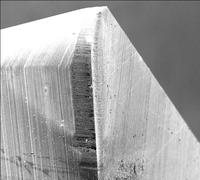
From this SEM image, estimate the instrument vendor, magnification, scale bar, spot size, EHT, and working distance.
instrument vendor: FEI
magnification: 0.093 K X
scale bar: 500000 nm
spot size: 4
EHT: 25 kV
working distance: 22.5 mm